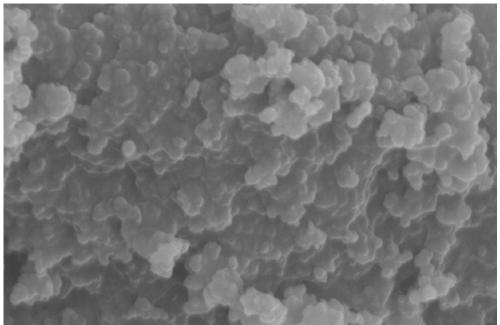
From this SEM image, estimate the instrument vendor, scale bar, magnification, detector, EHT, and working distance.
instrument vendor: Hitachi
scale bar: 1000 nm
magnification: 50 K X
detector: SE(U)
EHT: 10 kV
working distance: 8.7 mm